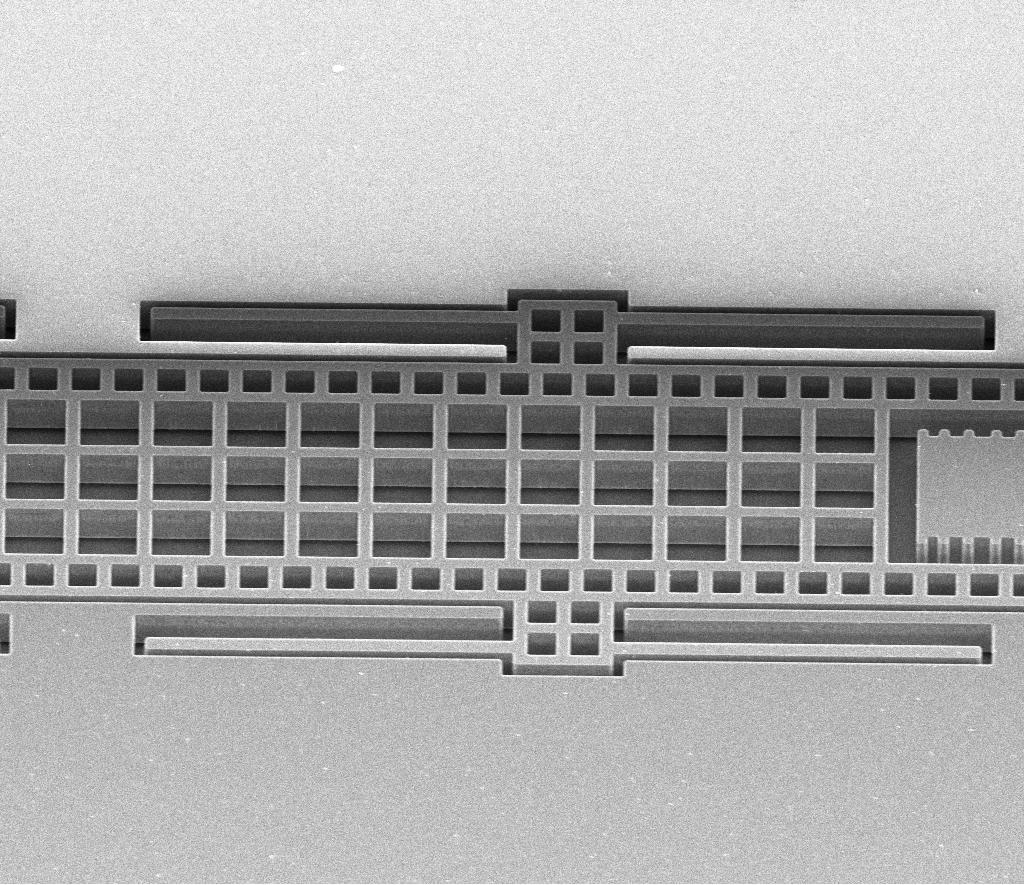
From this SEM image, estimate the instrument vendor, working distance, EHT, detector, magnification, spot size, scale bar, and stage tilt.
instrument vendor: FEI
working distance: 20.5 mm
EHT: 10 kV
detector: ETD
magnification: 0.75 K X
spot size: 2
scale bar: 100000 nm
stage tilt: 41°